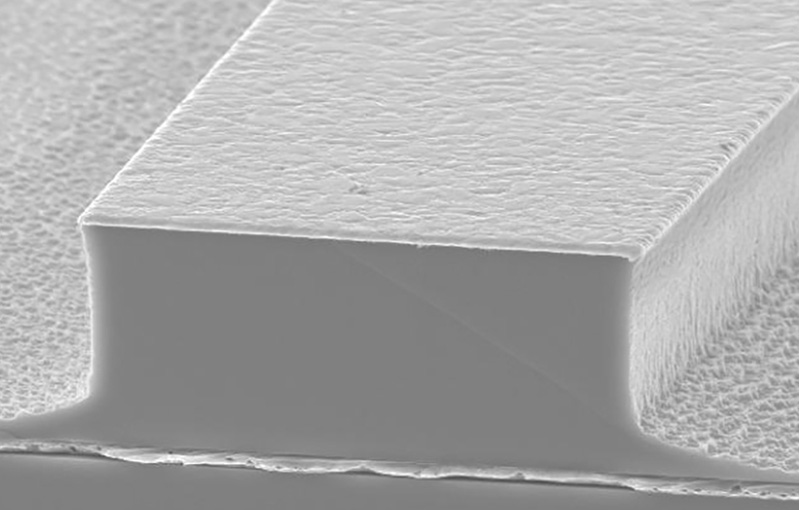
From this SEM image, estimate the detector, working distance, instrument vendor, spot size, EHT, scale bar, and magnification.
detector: SE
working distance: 15.9 mm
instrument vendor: FEI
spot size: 5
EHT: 15 kV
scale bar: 10000 nm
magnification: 3.5 K X